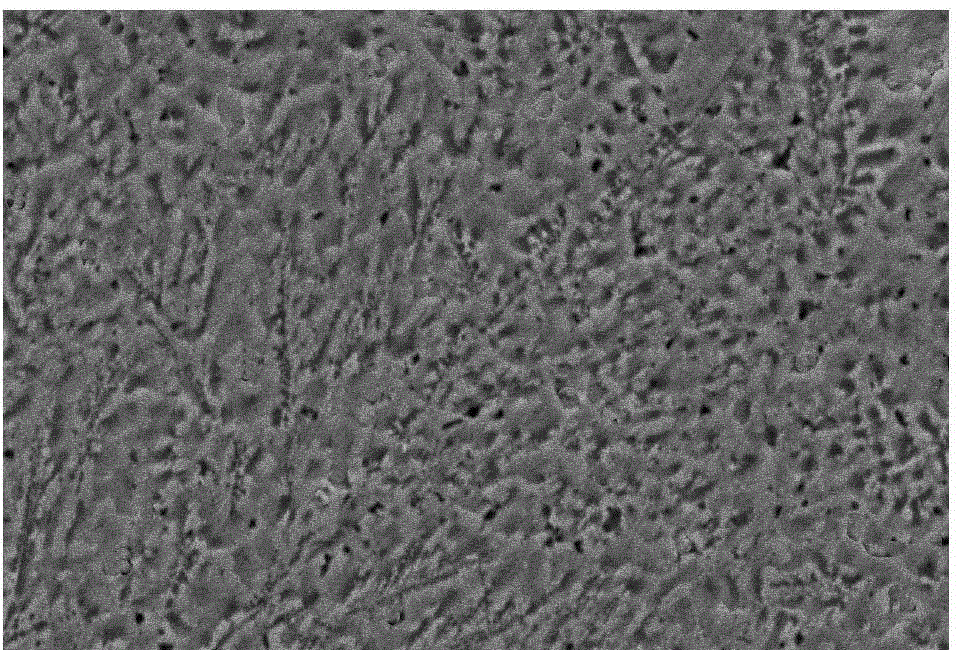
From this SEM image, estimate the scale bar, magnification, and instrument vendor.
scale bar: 5000 nm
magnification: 4 K X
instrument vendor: JEOL